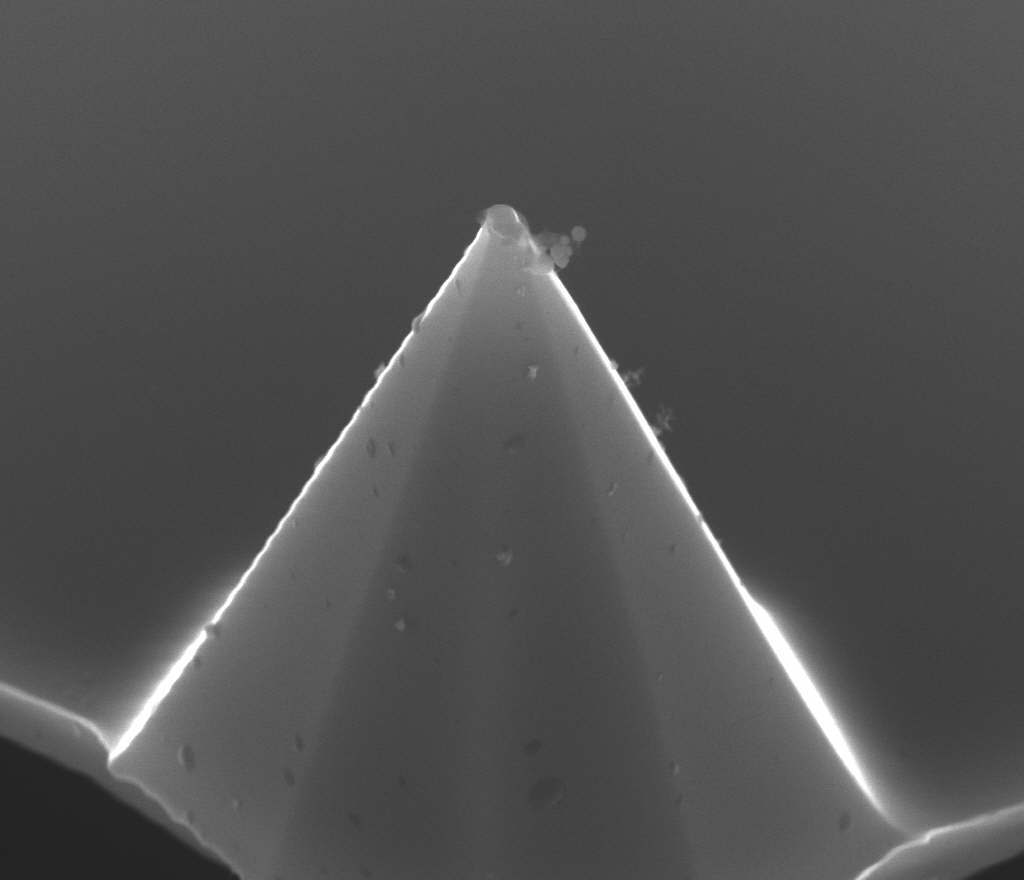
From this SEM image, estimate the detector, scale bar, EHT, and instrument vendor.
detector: HELIX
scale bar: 2000 nm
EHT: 15 kV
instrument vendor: FEI